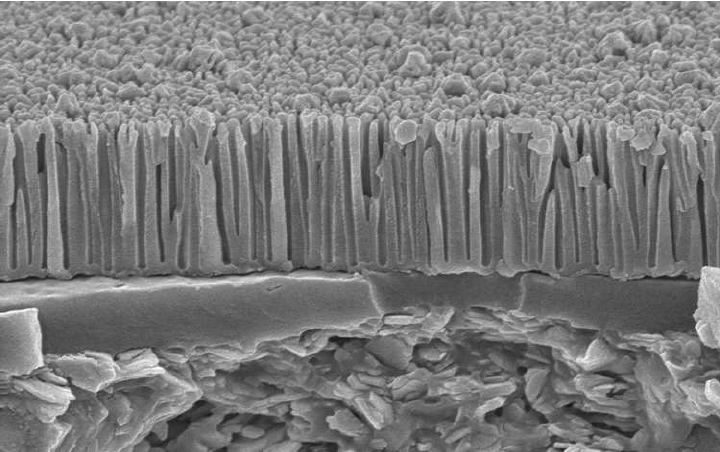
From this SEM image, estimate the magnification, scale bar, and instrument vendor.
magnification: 15 K X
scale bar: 2000 nm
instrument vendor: Hitachi